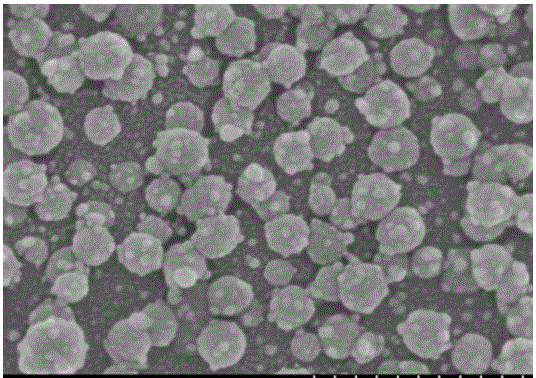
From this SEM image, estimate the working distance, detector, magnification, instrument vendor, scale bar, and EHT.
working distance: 8.3 mm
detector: SE(M)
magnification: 100 K X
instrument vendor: Hitachi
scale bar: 500 nm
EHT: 3 kV